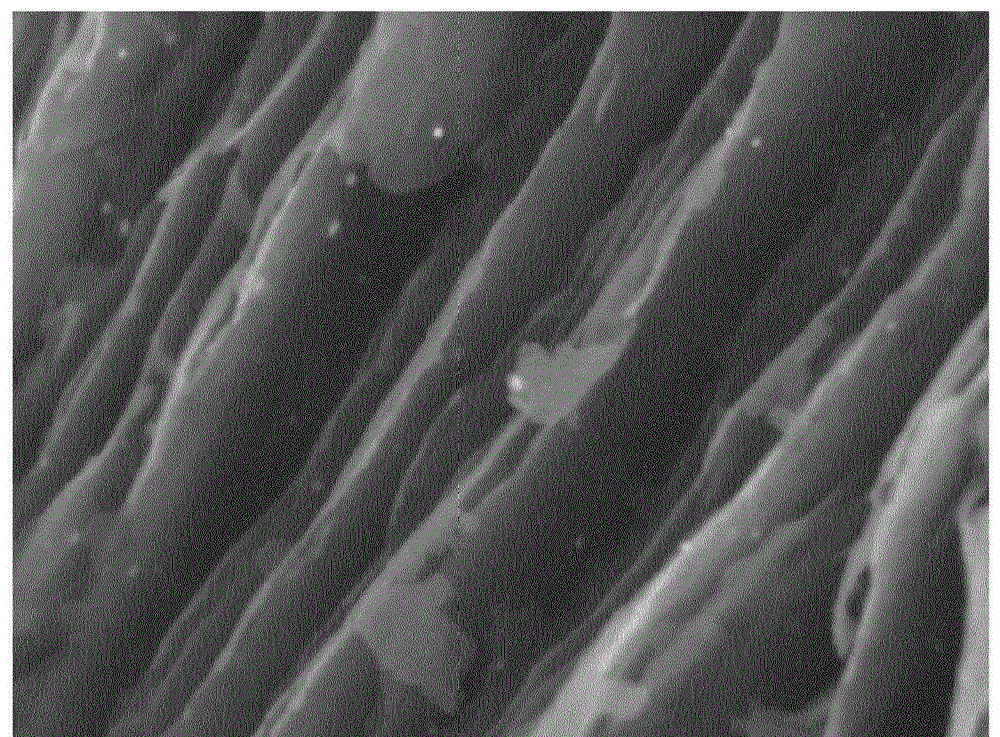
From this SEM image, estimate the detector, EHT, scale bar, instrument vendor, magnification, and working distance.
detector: COMPO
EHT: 5 kV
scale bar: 100 nm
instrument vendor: JEOL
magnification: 50 K X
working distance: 6 mm